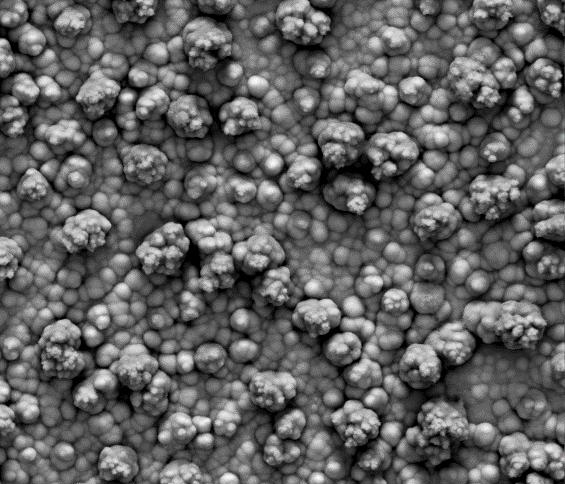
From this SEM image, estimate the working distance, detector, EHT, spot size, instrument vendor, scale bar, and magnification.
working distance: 10.4 mm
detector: Mix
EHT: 30 kV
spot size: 5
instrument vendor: FEI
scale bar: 50000 nm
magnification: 1 K X